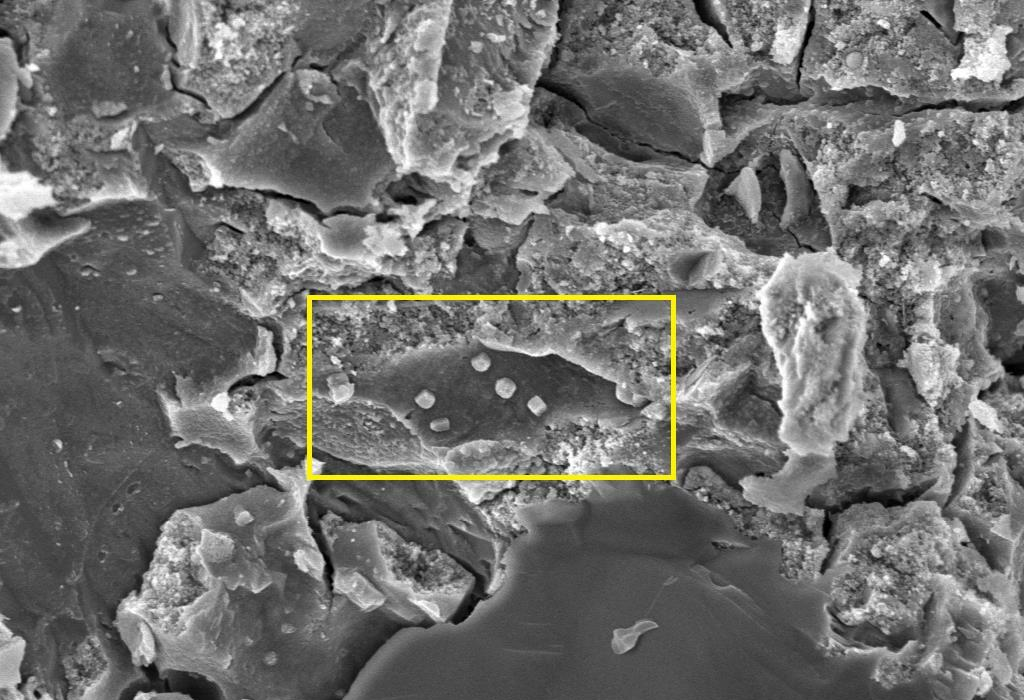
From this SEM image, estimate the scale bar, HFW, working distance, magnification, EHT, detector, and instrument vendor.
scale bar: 2000 nm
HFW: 59.47 µm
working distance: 12 mm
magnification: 5 K X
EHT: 10 kV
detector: SE1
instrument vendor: Zeiss